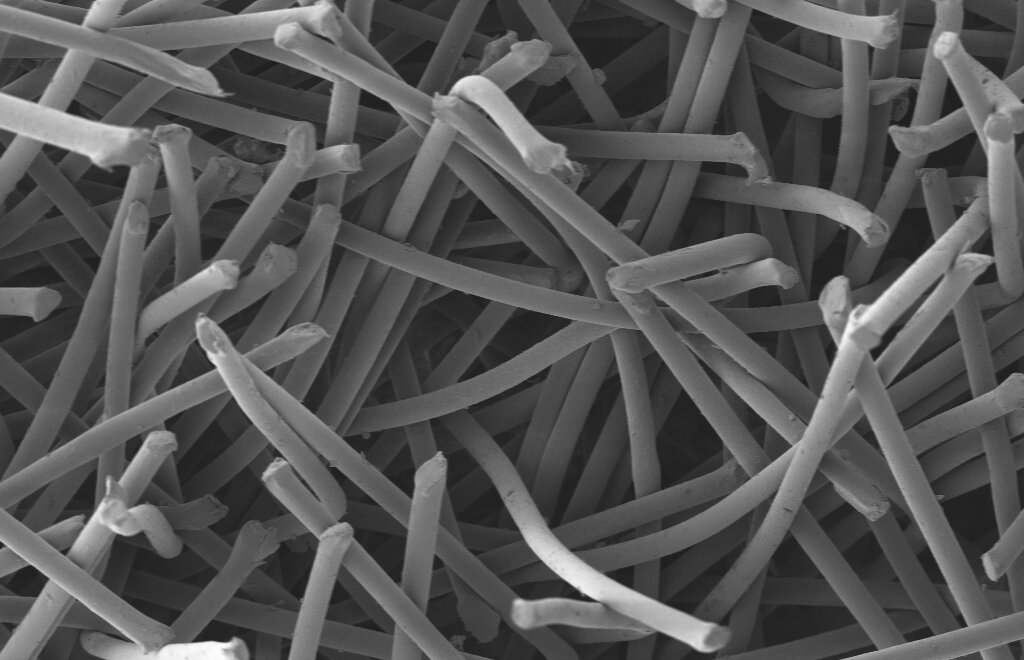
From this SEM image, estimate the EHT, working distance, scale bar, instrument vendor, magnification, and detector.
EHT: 2 kV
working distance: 13.6 mm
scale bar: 20000 nm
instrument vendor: Zeiss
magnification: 0.3 K X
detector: SE2